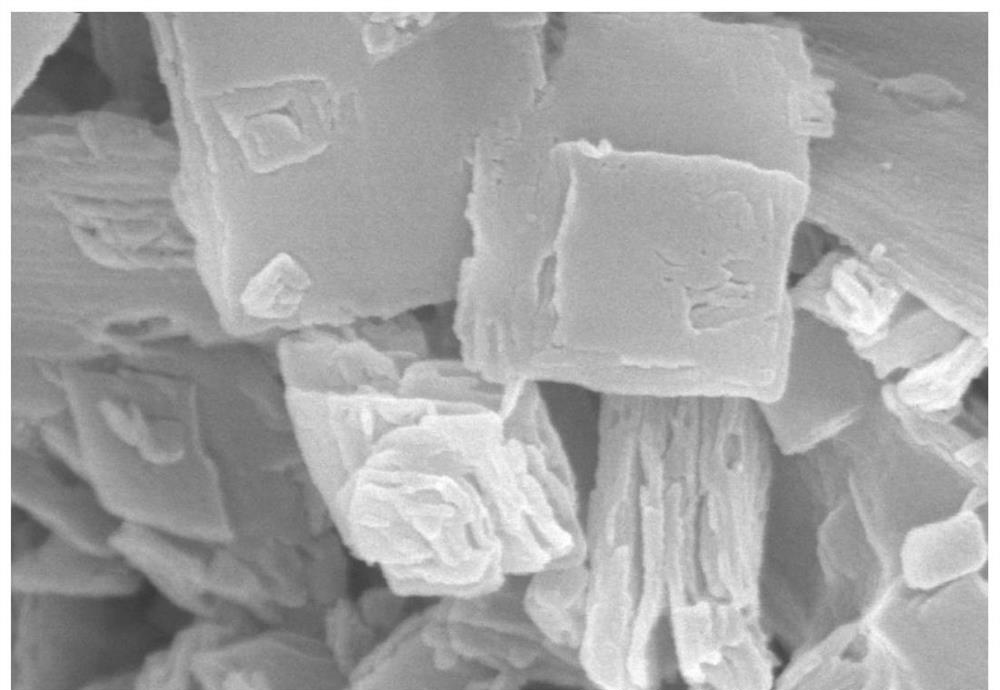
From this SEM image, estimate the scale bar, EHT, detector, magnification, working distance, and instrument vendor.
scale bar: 1000 nm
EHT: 15 kV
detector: SE(M)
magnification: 50 K X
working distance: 10.9 mm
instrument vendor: Hitachi